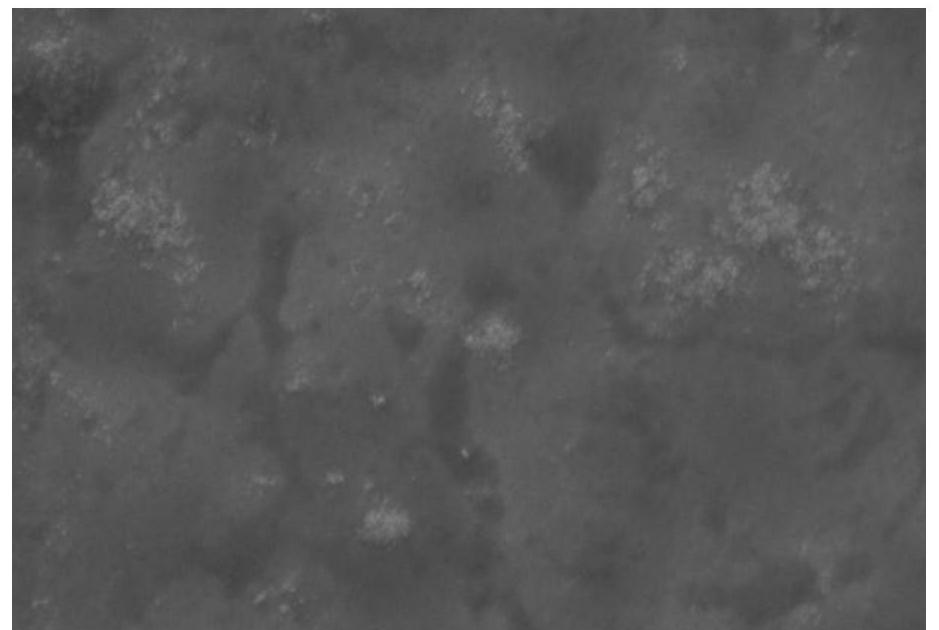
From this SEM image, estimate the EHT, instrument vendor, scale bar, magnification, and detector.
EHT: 20 kV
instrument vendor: JEOL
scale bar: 10000 nm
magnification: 1 K X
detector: SEI